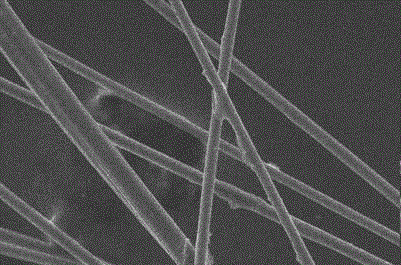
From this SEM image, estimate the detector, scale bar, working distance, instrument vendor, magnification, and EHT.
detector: SE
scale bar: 50000 nm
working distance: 10.5 mm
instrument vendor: FEI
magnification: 0.5 K X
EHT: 20 kV